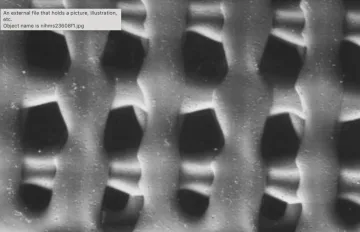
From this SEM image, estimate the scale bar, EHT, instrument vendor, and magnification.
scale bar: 2000000 nm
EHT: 25 kV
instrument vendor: JEOL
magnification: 0.025 K X